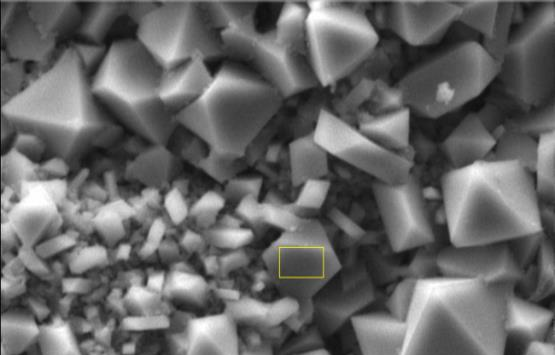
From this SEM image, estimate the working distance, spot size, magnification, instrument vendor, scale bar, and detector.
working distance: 11.1 mm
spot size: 4.1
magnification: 2 K X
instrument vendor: FEI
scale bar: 10000 nm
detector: SE